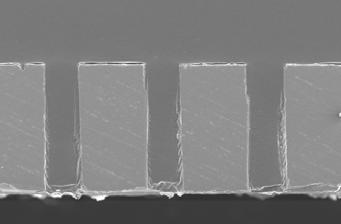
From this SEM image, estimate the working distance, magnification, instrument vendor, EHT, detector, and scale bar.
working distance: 15.6 mm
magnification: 0.15 K X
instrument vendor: JEOL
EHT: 15 kV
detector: SE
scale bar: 300000 nm